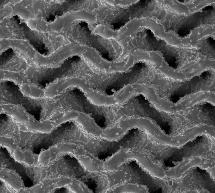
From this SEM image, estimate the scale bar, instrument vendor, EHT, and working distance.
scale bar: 1000000 nm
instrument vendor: Tescan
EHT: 20 kV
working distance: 25.06 mm